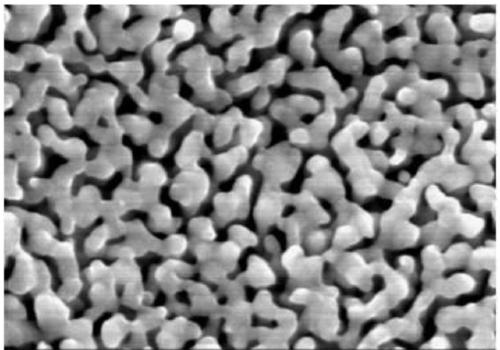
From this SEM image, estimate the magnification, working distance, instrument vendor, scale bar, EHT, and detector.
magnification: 100 K X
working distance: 8.3 mm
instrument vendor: Hitachi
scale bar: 500 nm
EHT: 15 kV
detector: SE(M)